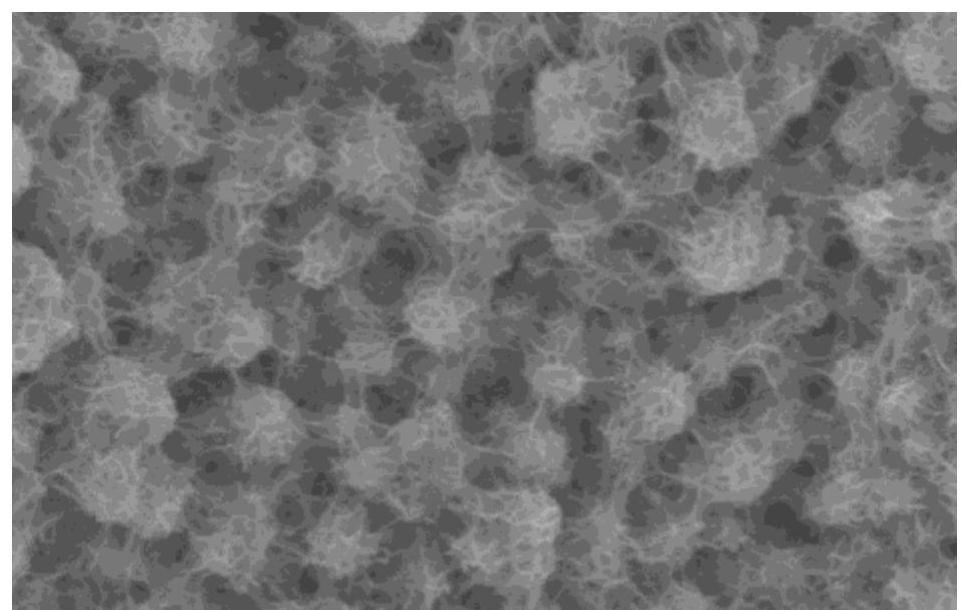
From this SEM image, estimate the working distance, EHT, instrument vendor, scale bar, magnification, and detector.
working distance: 16.7 mm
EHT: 5 kV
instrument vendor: Hitachi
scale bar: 1000 nm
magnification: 35 K X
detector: SE(U)